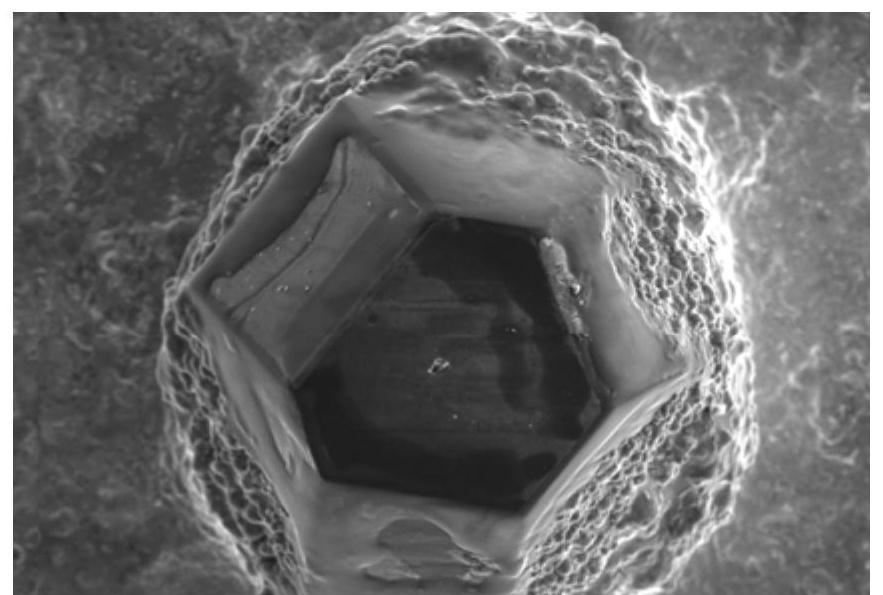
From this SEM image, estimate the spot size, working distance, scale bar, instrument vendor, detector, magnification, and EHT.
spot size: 55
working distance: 12 mm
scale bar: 100000 nm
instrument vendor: JEOL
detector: SEI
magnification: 0.15 K X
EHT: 20 kV